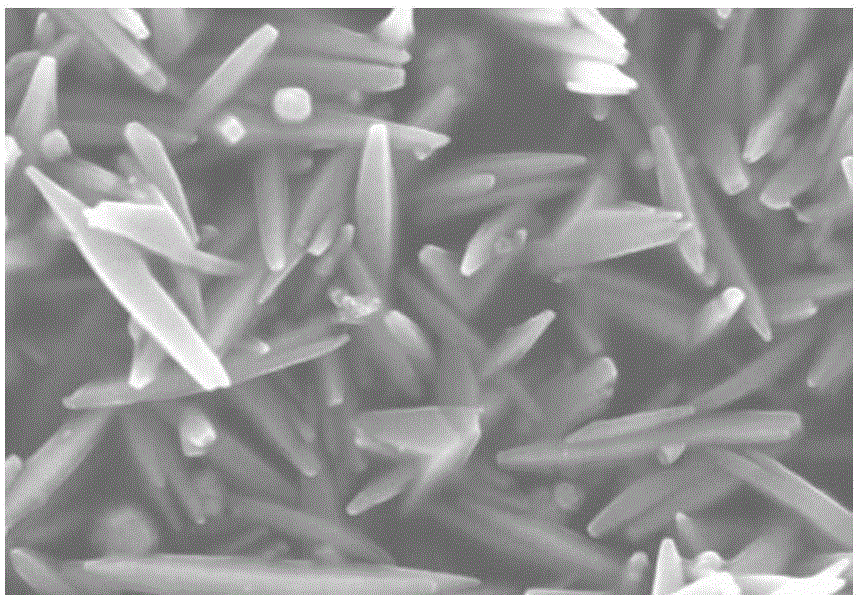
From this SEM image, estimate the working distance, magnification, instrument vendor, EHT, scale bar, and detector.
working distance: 5 mm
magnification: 50 K X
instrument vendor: Hitachi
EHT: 5 kV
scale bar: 1000 nm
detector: SE(U)